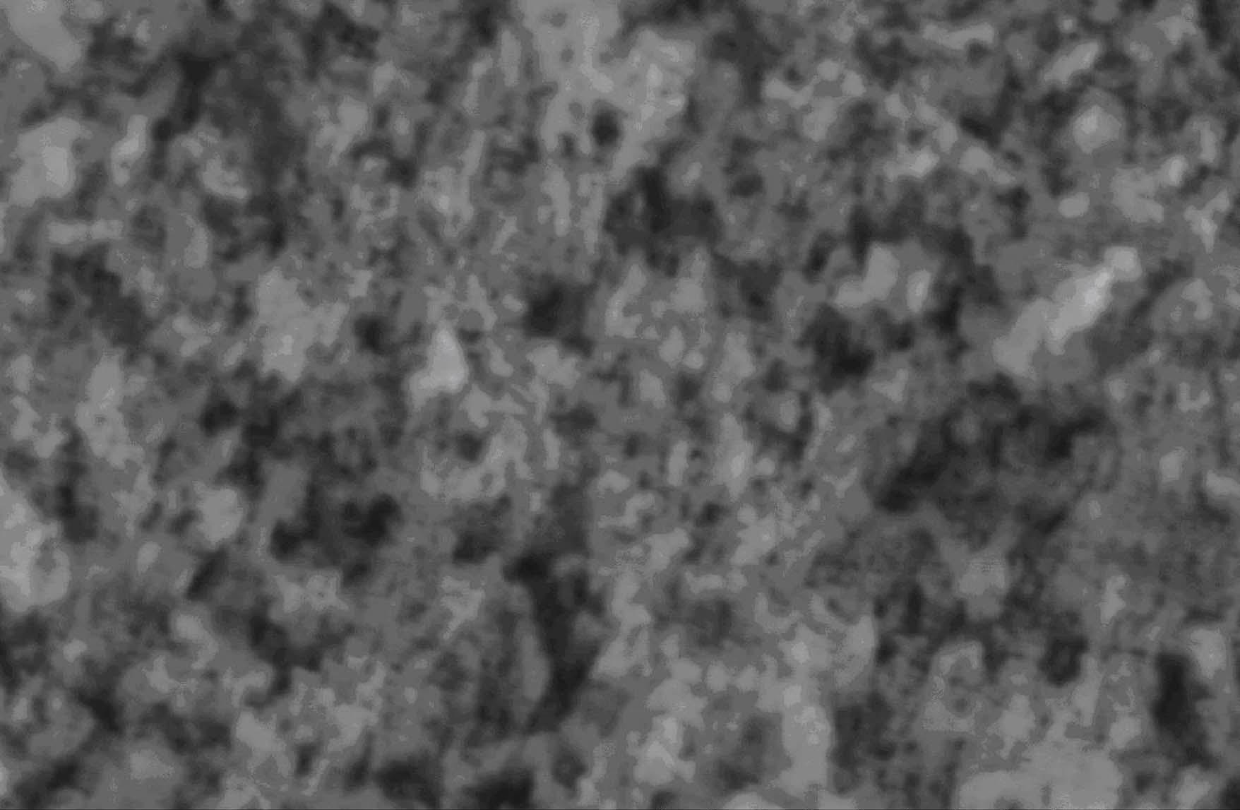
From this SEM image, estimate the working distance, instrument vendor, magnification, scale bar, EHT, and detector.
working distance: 5.4 mm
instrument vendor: Hitachi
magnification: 10 K X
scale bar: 5000 nm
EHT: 10 kV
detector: SE